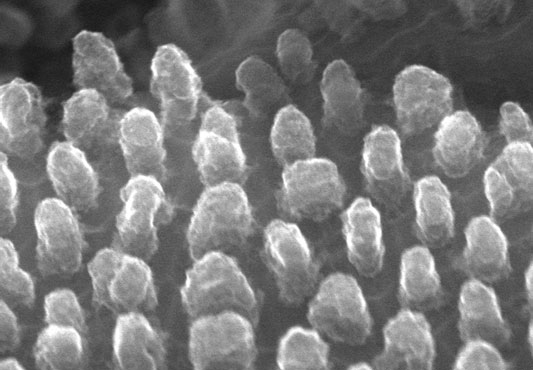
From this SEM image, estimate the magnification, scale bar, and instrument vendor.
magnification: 200 K X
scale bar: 200 nm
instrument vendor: Hitachi